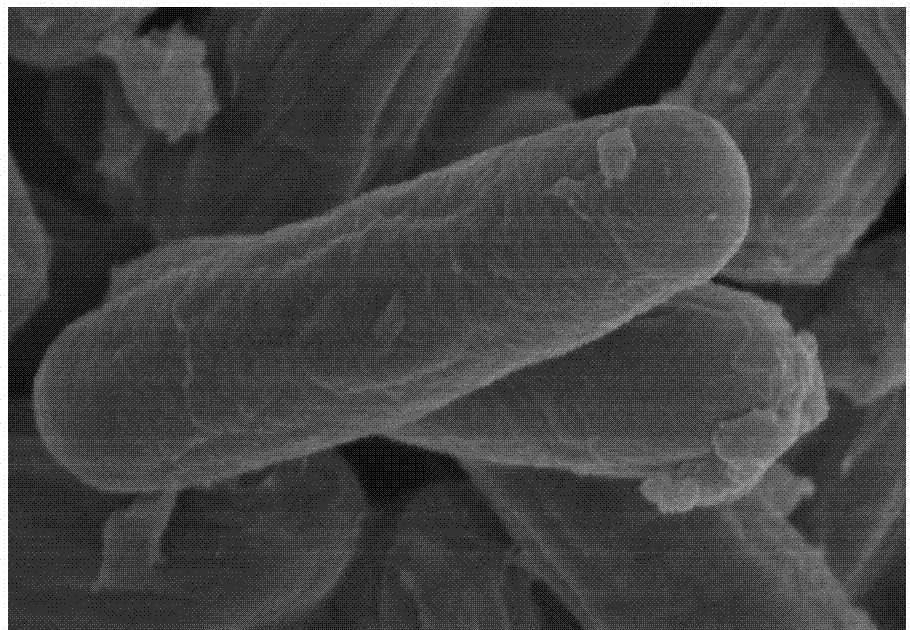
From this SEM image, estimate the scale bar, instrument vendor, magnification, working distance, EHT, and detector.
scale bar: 1000 nm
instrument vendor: Hitachi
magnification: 50 K X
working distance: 9.6 mm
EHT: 3 kV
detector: SE(UL)